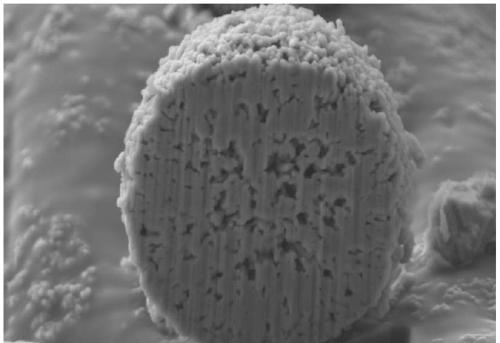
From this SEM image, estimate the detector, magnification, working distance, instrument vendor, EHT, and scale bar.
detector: SE2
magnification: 10 K X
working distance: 5.2 mm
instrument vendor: Zeiss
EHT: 5 kV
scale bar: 1000 nm